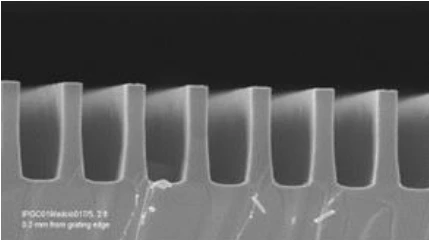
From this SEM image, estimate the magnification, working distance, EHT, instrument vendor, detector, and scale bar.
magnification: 50 K X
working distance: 2 mm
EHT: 5 kV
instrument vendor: Zeiss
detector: InLens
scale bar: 1000 nm